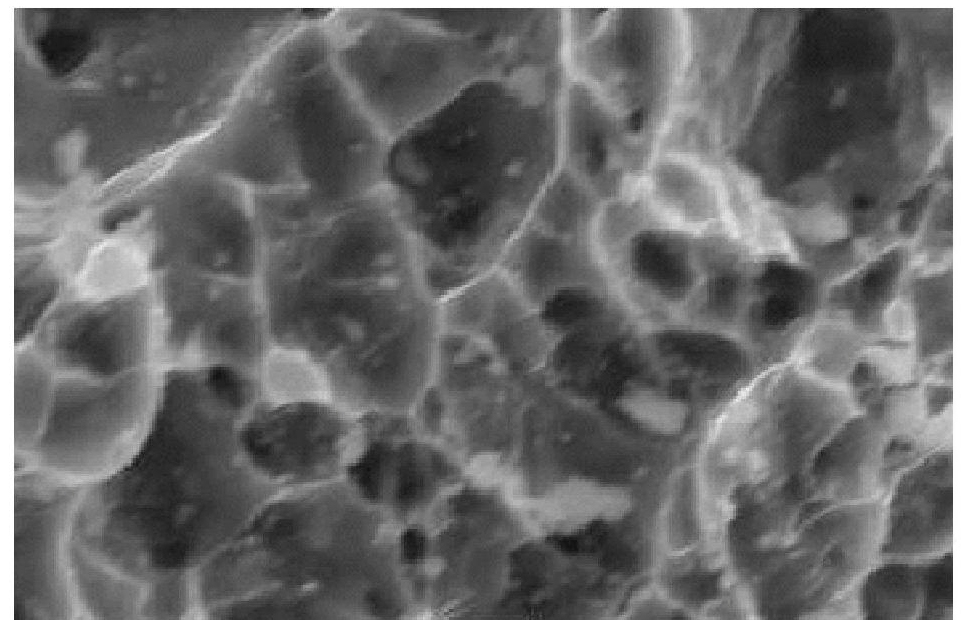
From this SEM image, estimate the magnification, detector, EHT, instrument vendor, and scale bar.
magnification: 3.5 K X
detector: SEI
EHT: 25 kV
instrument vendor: JEOL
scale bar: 5000 nm